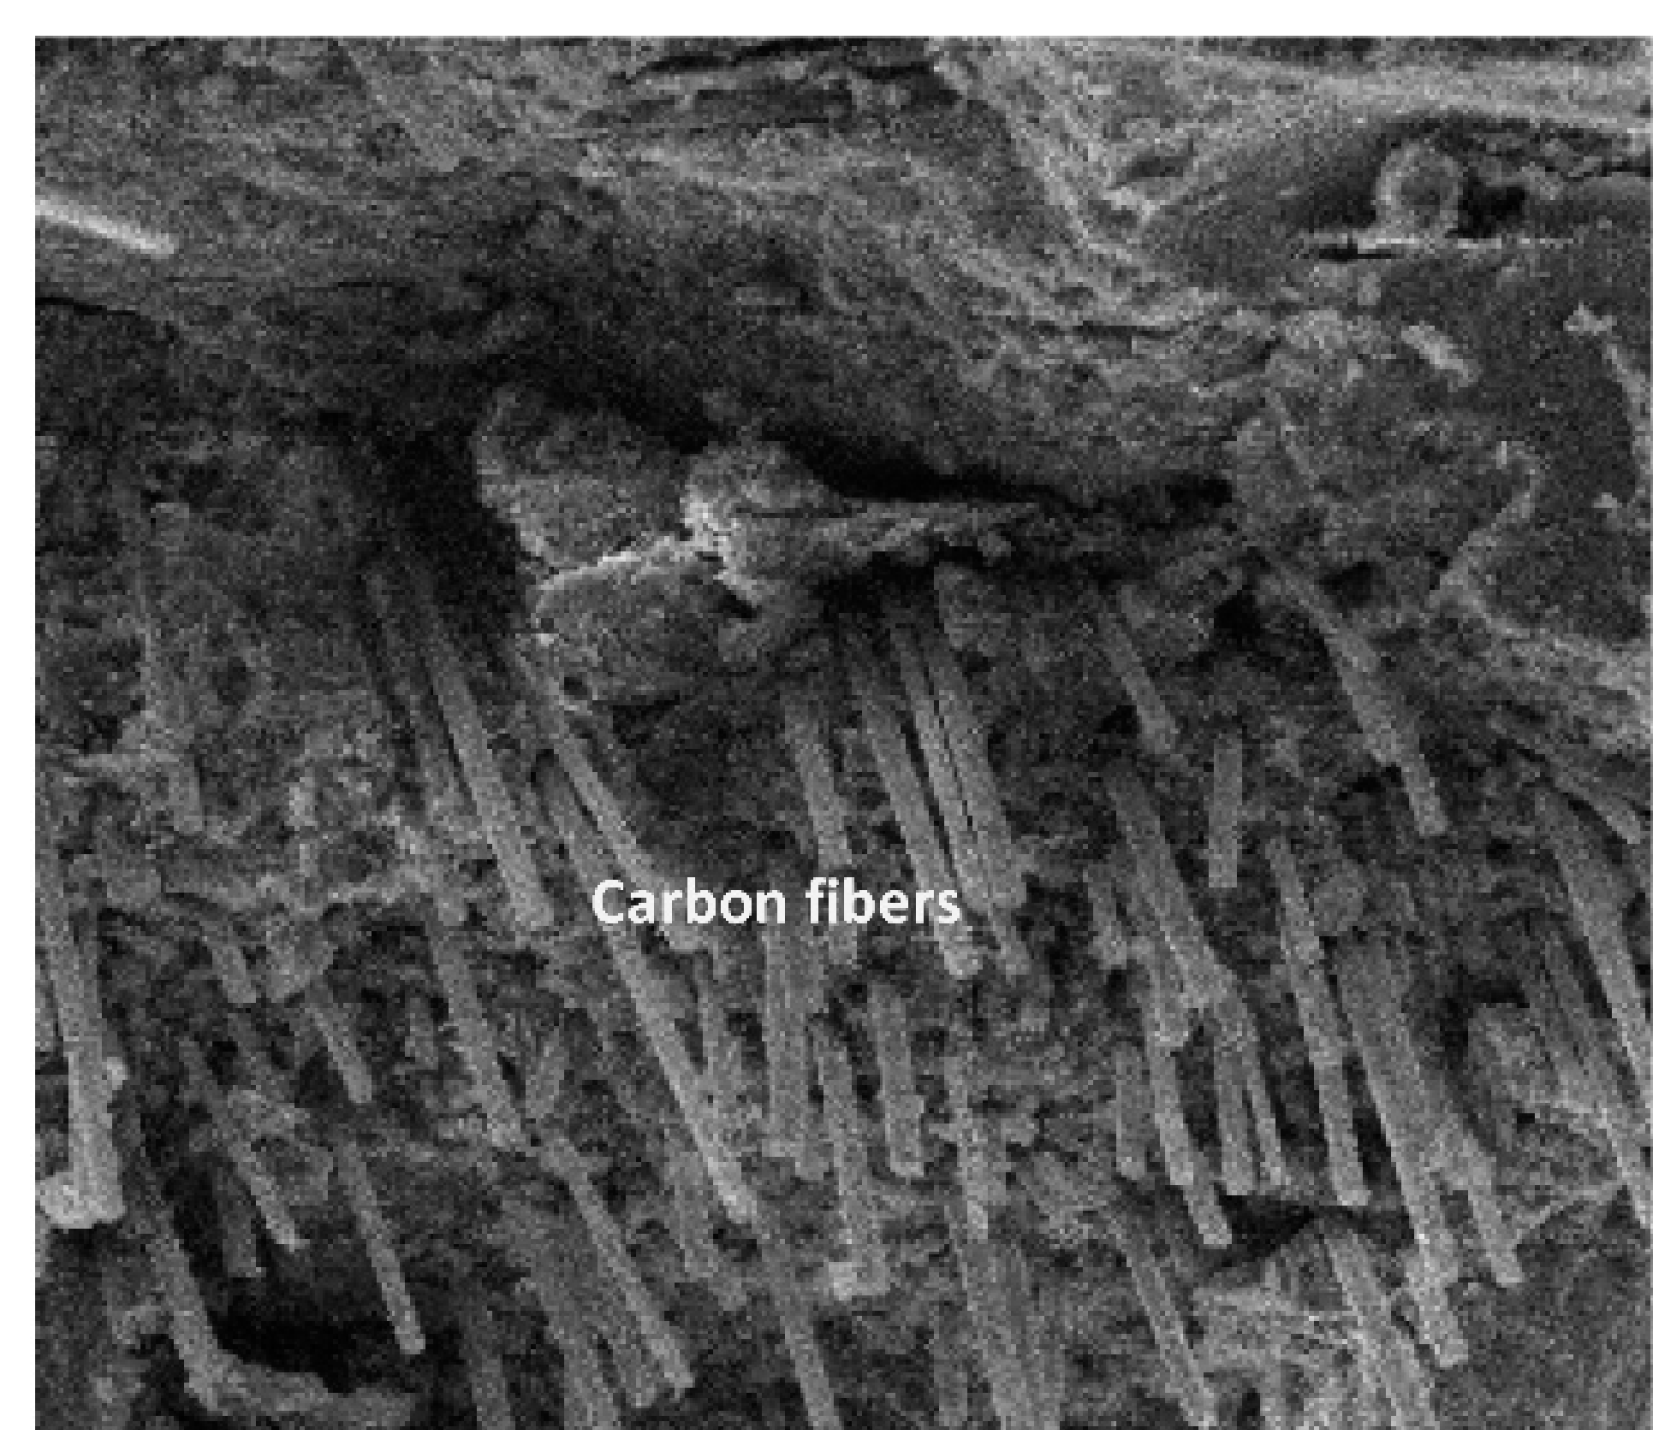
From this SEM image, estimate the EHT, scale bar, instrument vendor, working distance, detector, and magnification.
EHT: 30 kV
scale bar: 200000 nm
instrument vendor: FEI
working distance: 21.3 mm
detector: ETD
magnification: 0.5 K X